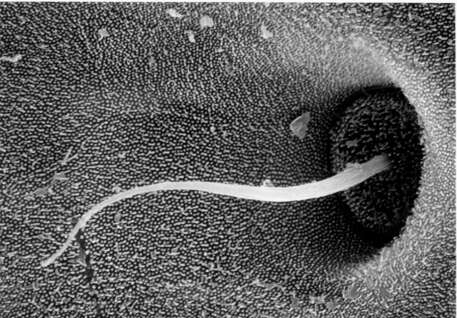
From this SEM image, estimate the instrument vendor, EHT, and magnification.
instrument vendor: JEOL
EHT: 20 kV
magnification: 3.2 K X